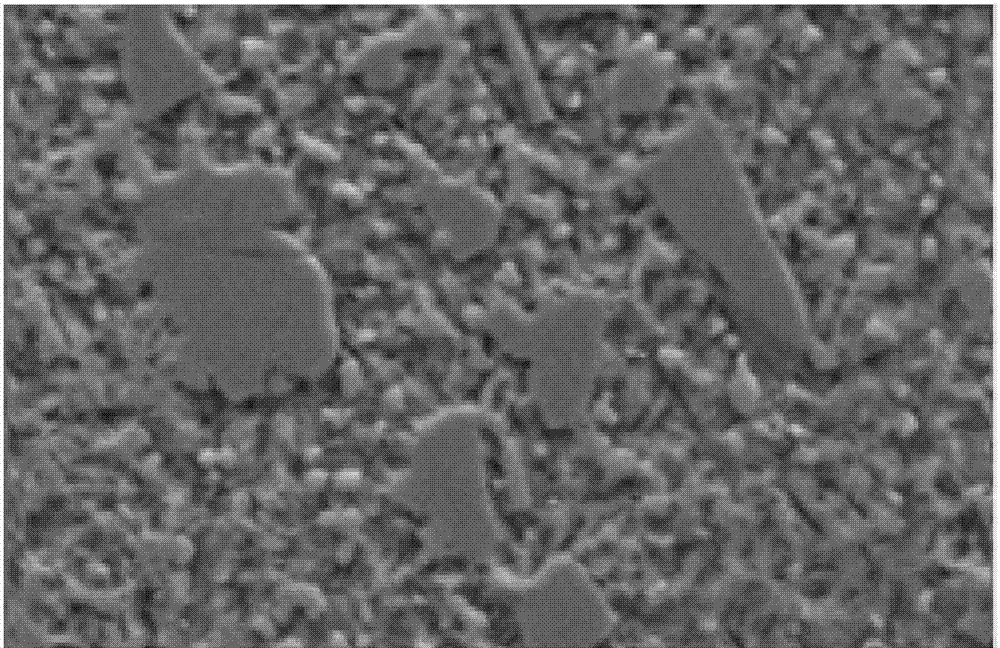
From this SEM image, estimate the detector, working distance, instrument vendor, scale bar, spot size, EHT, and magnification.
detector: SE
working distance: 7.9 mm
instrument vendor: FEI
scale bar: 10000 nm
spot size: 3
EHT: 20 kV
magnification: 5 K X